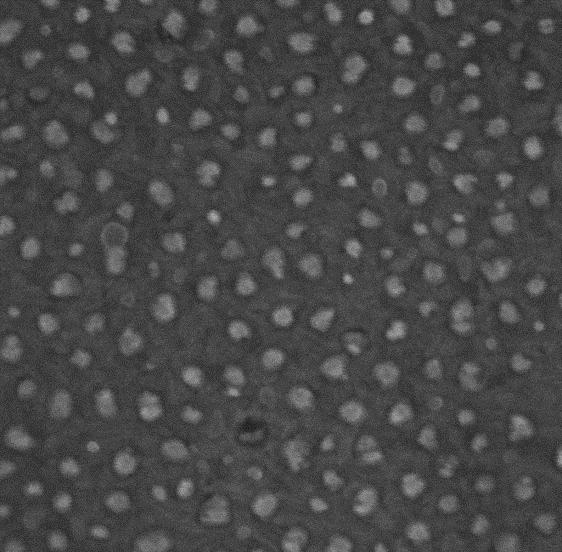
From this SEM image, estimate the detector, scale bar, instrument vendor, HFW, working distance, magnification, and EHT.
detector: InBeam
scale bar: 200 nm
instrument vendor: Tescan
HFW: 1.156 µm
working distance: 3.001 mm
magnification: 250 K X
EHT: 15 kV